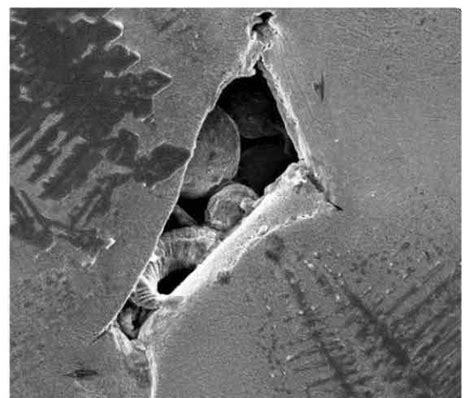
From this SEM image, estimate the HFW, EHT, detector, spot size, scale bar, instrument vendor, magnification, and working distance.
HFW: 149 µm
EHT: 15 kV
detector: ETD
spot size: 4.9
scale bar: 50000 nm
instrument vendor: FEI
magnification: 2 K X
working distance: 11.9 mm